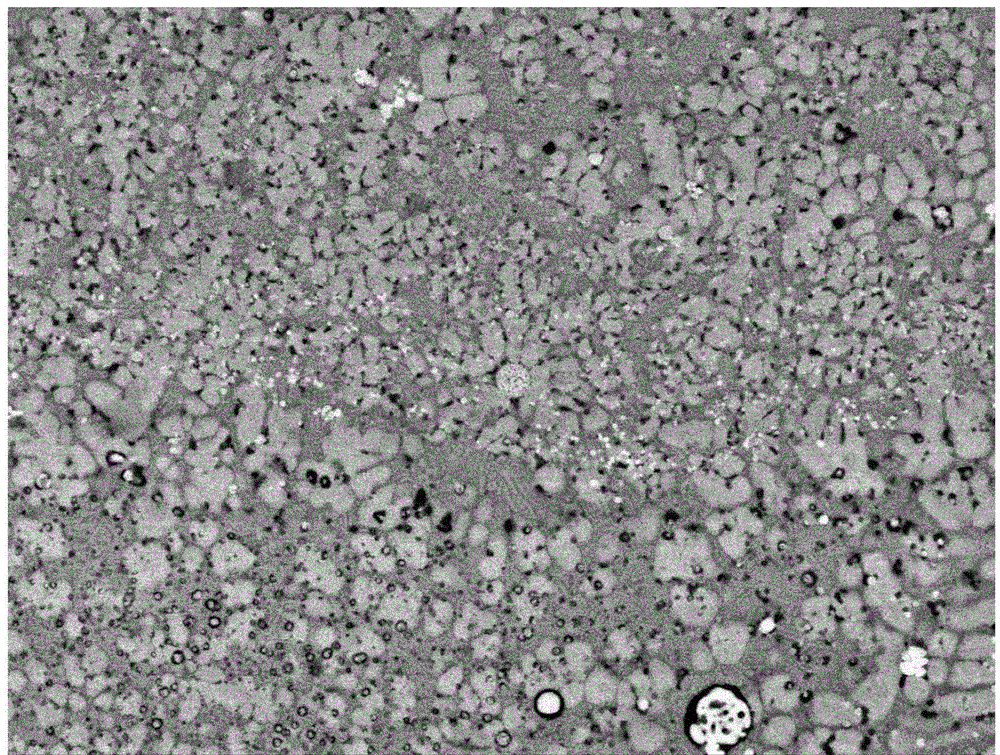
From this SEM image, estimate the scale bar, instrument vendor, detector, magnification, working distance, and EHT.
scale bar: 1000 nm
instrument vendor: JEOL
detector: COMPO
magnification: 5 K X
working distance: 7.7 mm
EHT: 15 kV